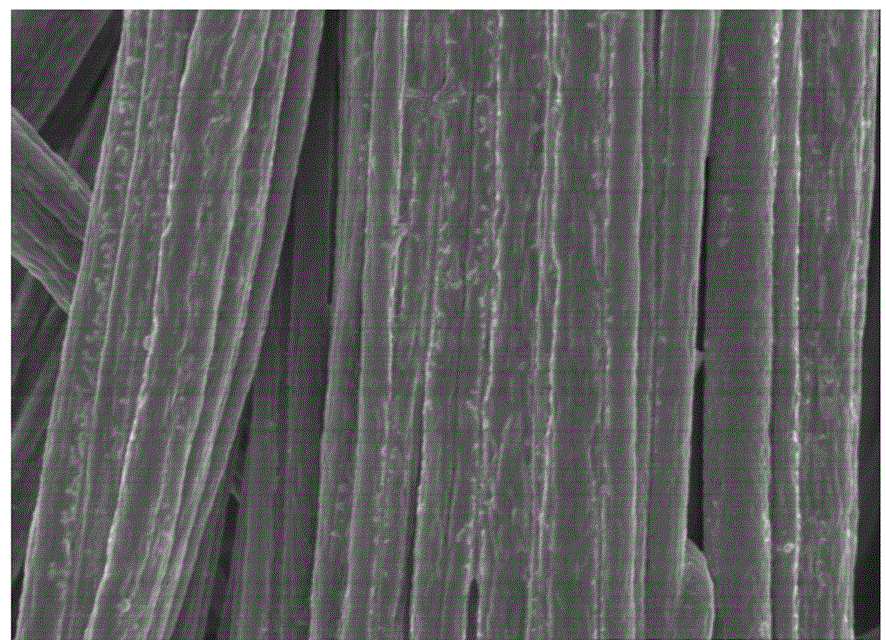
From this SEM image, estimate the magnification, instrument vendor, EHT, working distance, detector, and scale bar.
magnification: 20 K X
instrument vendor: Hitachi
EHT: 3 kV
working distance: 8.8 mm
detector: SE(U)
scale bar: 2000 nm